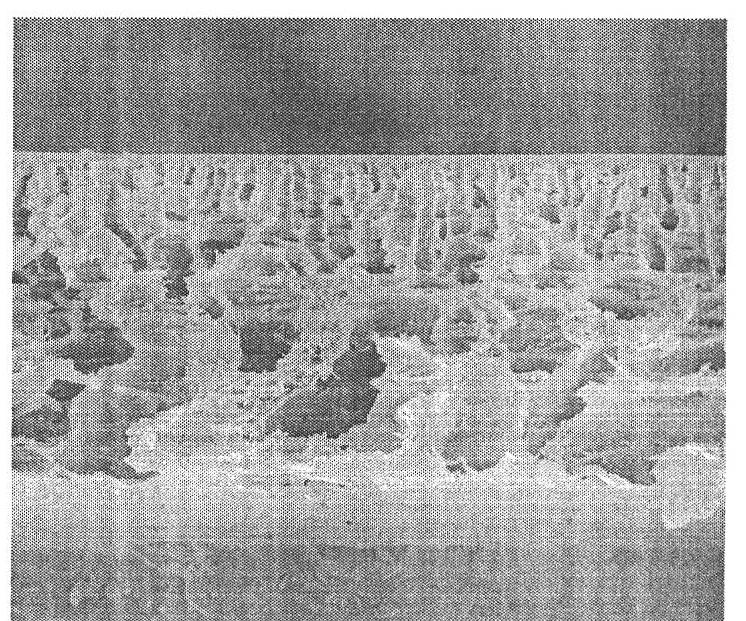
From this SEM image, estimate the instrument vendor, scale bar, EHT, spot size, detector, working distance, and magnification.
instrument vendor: FEI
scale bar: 50000 nm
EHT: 10 kV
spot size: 3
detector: ETD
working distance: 5.4 mm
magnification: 1 K X